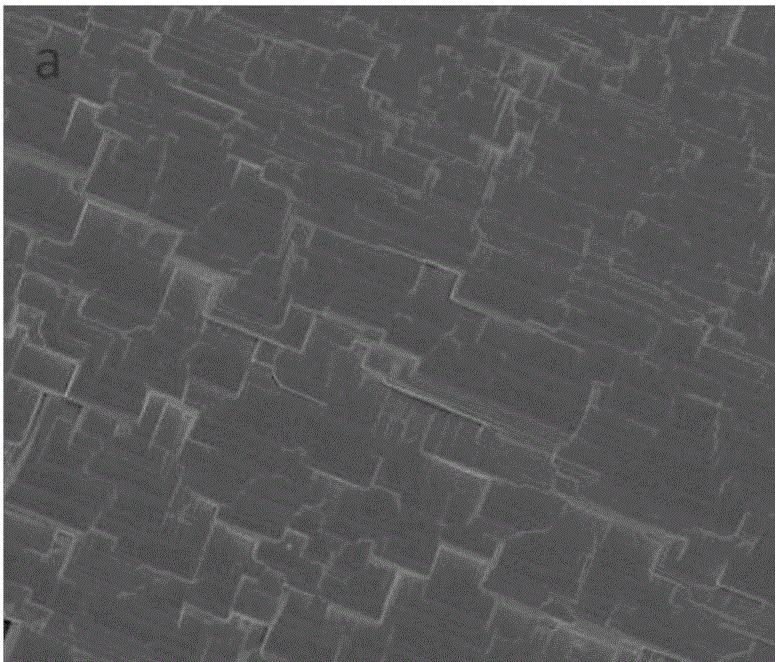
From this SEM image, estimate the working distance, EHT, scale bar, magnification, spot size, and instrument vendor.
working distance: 5.4 mm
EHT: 3 kV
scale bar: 4000 nm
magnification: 20 K X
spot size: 3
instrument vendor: FEI